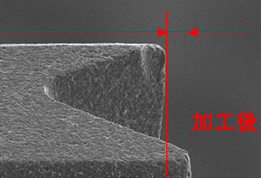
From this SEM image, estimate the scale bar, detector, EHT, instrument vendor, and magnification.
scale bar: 10000 nm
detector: SEI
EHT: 10 kV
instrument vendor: JEOL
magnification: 1.5 K X